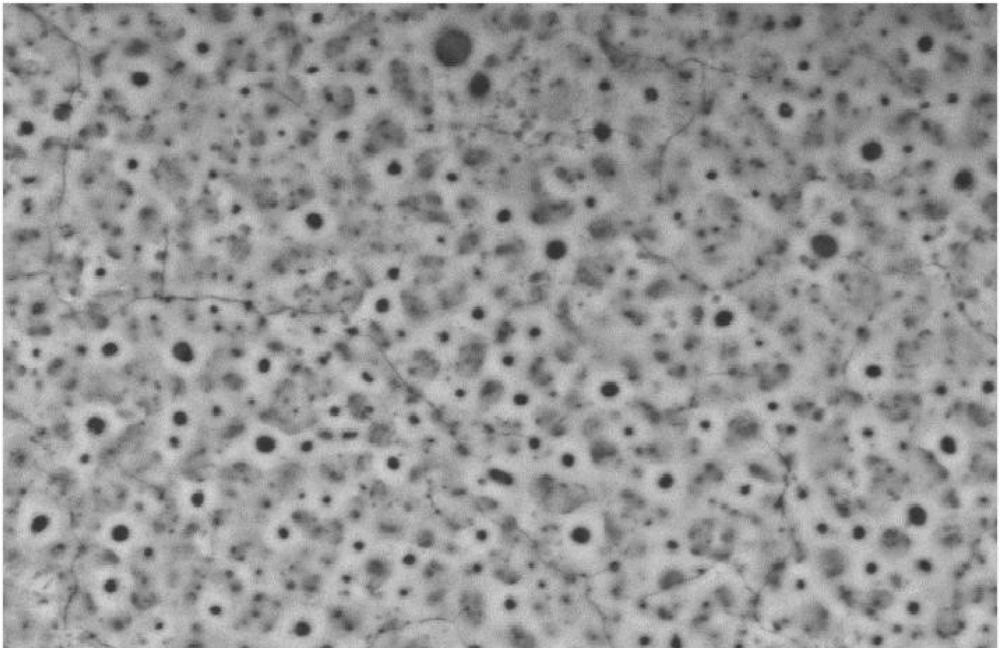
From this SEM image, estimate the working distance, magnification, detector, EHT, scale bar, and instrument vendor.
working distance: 10.8 mm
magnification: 1 K X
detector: BSE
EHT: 25 kV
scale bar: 10000 nm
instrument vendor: FEI